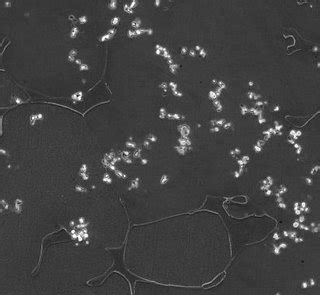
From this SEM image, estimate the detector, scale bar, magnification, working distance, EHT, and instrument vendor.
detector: SEI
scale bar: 10000 nm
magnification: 1 K X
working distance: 10 mm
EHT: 15 kV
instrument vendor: JEOL